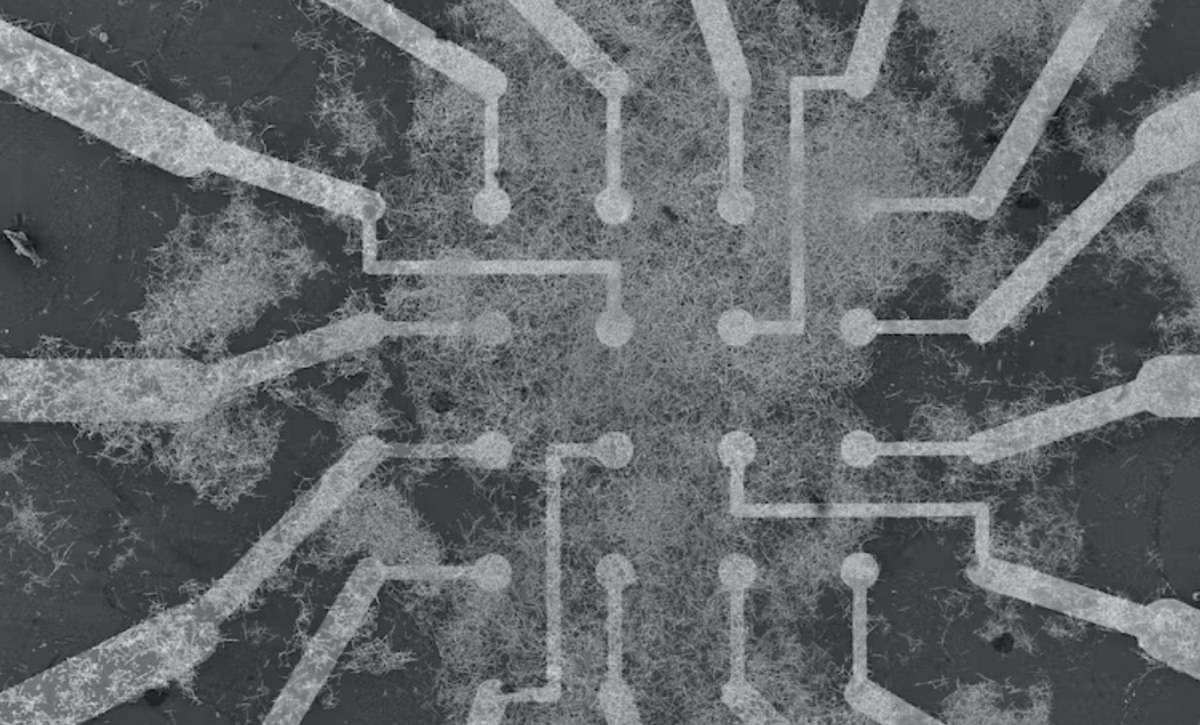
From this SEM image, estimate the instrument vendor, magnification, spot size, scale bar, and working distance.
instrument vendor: Hitachi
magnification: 0.065 K X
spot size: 50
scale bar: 200000 nm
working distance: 14 mm